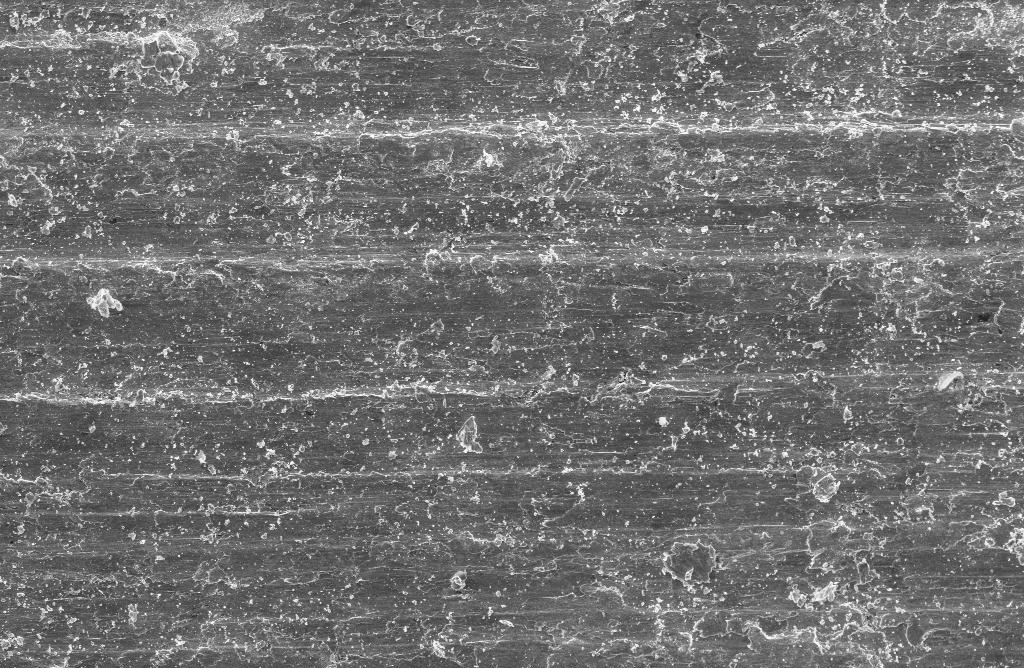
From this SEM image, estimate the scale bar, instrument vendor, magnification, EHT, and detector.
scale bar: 100000 nm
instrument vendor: Zeiss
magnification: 0.1 K X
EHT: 20 kV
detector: SE1